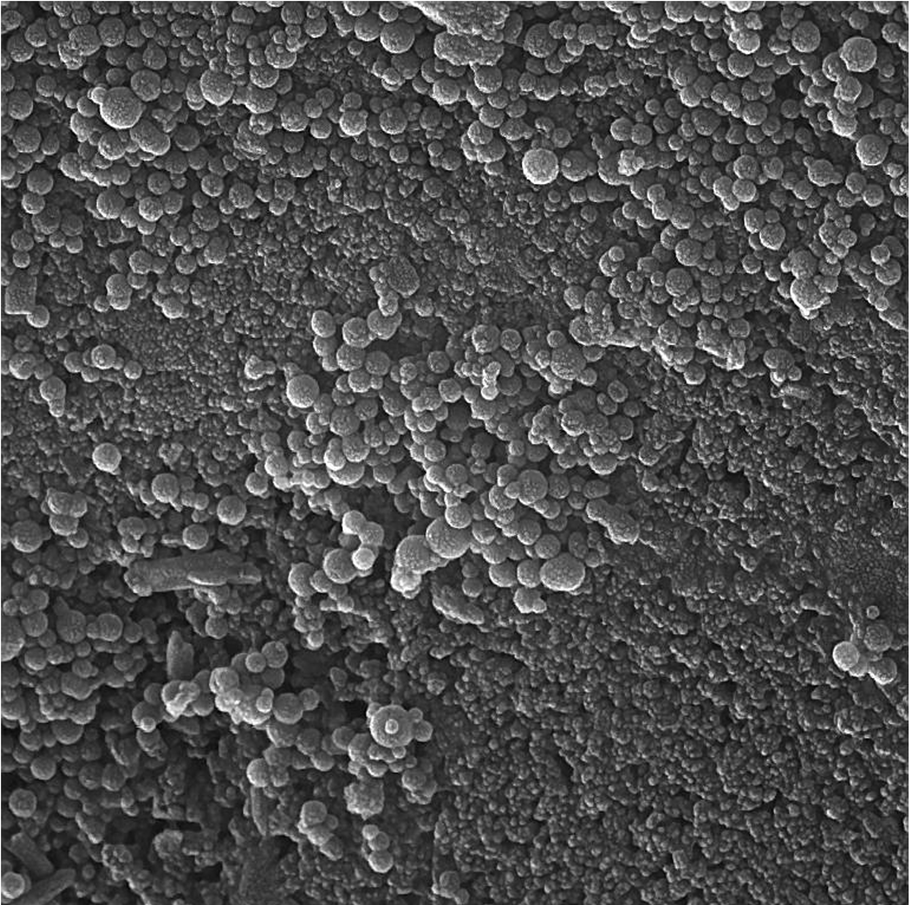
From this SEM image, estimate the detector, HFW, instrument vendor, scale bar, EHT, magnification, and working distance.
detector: In-Beam SE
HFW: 2.77 µm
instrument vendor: Tescan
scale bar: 500 nm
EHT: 15 kV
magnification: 75 K X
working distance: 4.94 mm